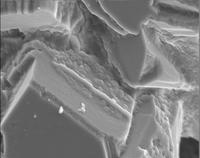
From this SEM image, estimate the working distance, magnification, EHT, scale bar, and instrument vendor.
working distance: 13 mm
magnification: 4.5 K X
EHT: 9 kV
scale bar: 1000 nm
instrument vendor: JEOL